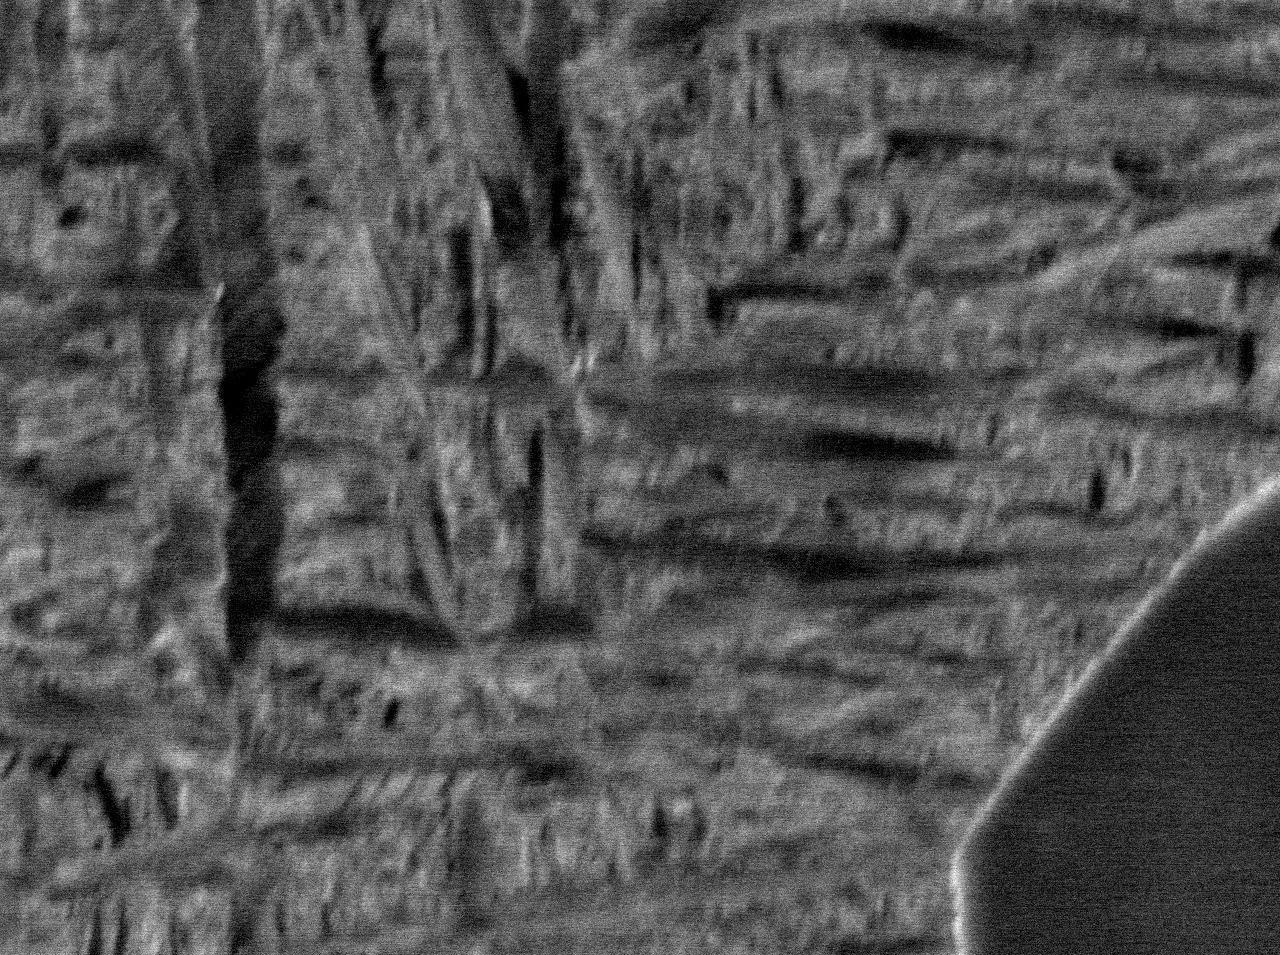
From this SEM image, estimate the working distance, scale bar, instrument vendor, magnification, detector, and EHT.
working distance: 10 mm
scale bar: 1000 nm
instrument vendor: JEOL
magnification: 10 K X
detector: COMPO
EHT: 15 kV